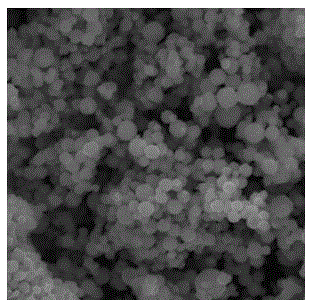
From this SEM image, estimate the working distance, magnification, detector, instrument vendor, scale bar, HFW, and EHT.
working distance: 9.3 mm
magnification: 6.13 K X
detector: SE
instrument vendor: Tescan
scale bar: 10000 nm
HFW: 33.8 µm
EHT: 30 kV